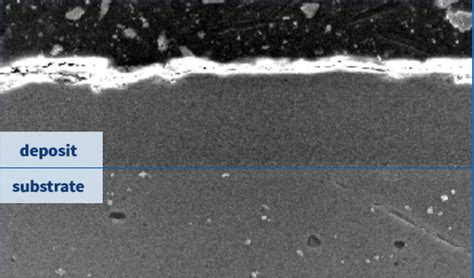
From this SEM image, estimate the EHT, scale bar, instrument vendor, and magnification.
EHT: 15 kV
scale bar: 10000 nm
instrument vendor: JEOL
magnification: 1 K X